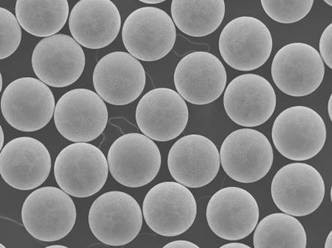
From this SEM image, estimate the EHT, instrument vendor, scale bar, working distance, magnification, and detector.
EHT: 10 kV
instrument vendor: JEOL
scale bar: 100000 nm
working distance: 9.6 mm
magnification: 0.1 K X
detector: SEI